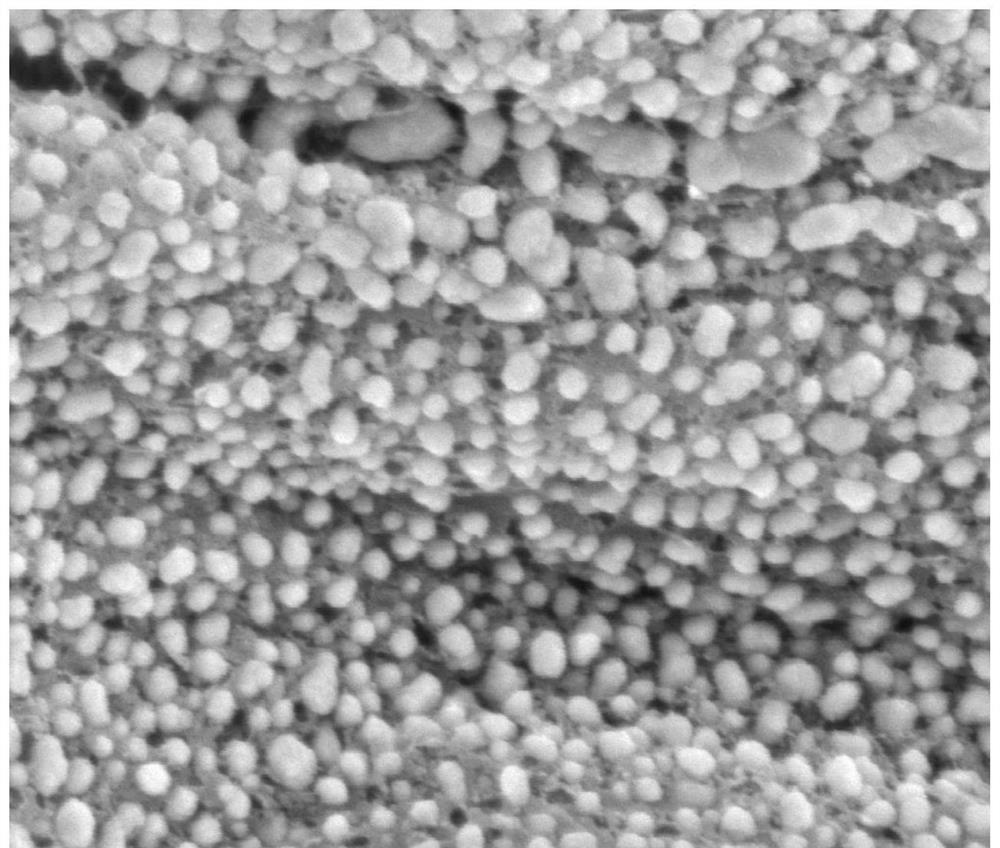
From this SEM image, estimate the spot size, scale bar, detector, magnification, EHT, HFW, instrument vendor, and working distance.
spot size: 4.5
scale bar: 500 nm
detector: SE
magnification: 120 K X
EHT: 5 kV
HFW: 2.49 µm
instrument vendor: FEI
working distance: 10.2 mm